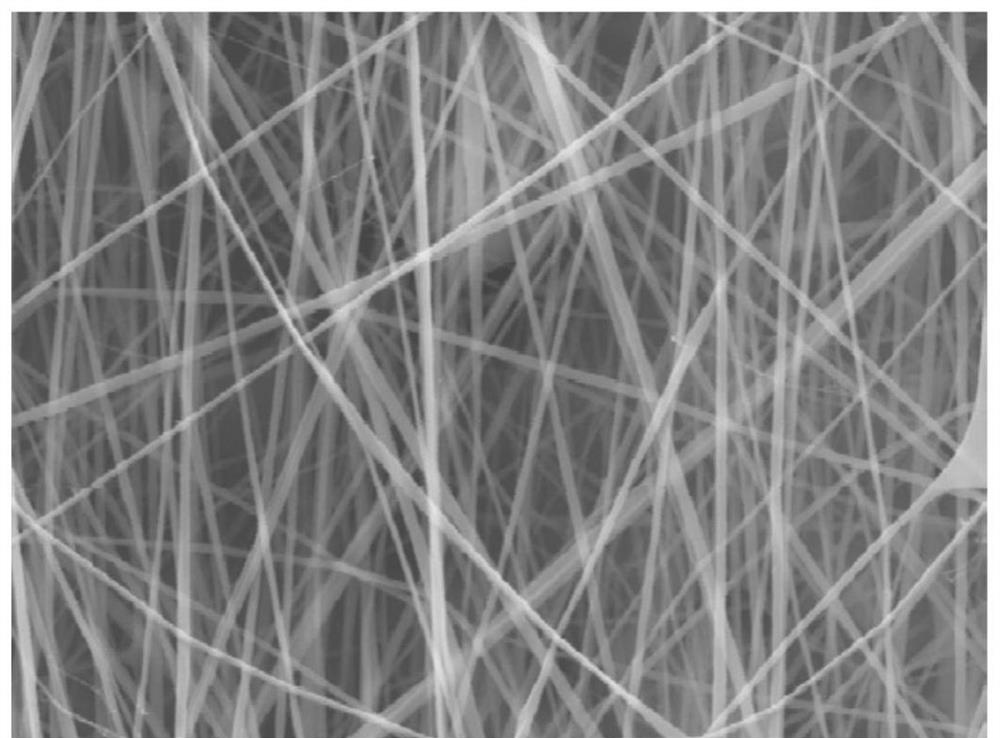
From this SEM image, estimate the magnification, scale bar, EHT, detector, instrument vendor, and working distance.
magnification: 6 K X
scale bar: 2000 nm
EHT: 15 kV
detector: SED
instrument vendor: JEOL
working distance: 12.6 mm